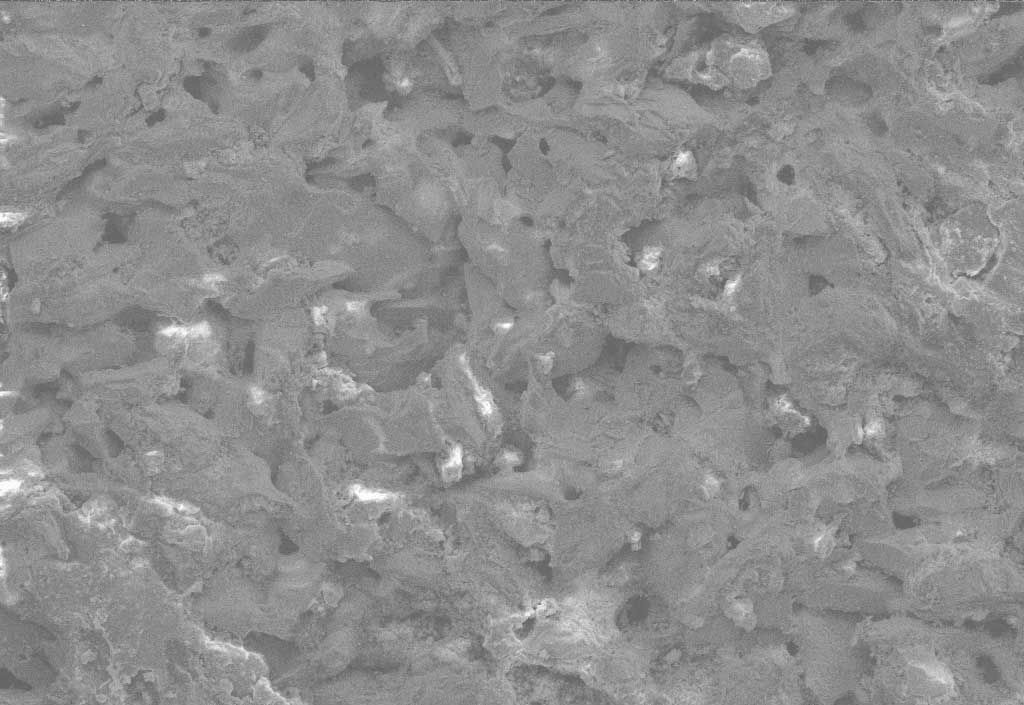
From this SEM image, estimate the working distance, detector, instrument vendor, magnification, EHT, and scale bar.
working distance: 43 mm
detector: SE1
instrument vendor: Zeiss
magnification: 0.08 K X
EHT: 20 kV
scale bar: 100000 nm